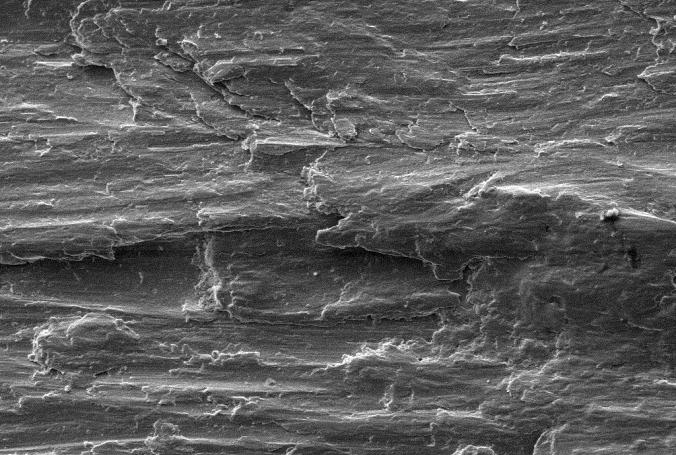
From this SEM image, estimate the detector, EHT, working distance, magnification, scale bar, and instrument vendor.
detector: SE1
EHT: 20 kV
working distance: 7.5 mm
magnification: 0.5 K X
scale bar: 20000 nm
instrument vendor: Zeiss